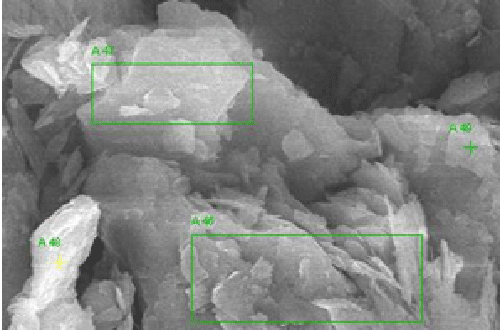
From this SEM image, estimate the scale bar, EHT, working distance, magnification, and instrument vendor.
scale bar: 3000 nm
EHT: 15 kV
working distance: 15.6 mm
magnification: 10 K X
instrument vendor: Tescan